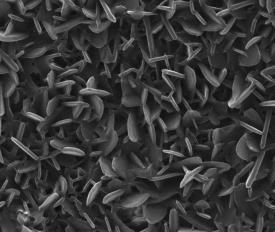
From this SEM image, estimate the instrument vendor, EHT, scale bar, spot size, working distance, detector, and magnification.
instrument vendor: FEI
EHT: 30 kV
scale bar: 2000 nm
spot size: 3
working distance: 9.1 mm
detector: ETD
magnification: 50 K X